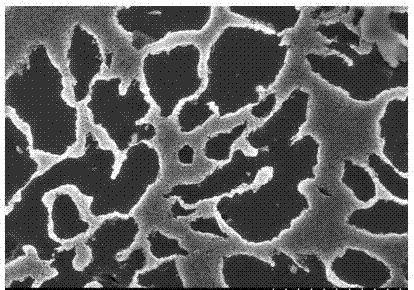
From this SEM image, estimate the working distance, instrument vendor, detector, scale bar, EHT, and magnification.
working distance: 7 mm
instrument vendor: Hitachi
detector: SE(M)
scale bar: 20000 nm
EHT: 15 kV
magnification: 2 K X